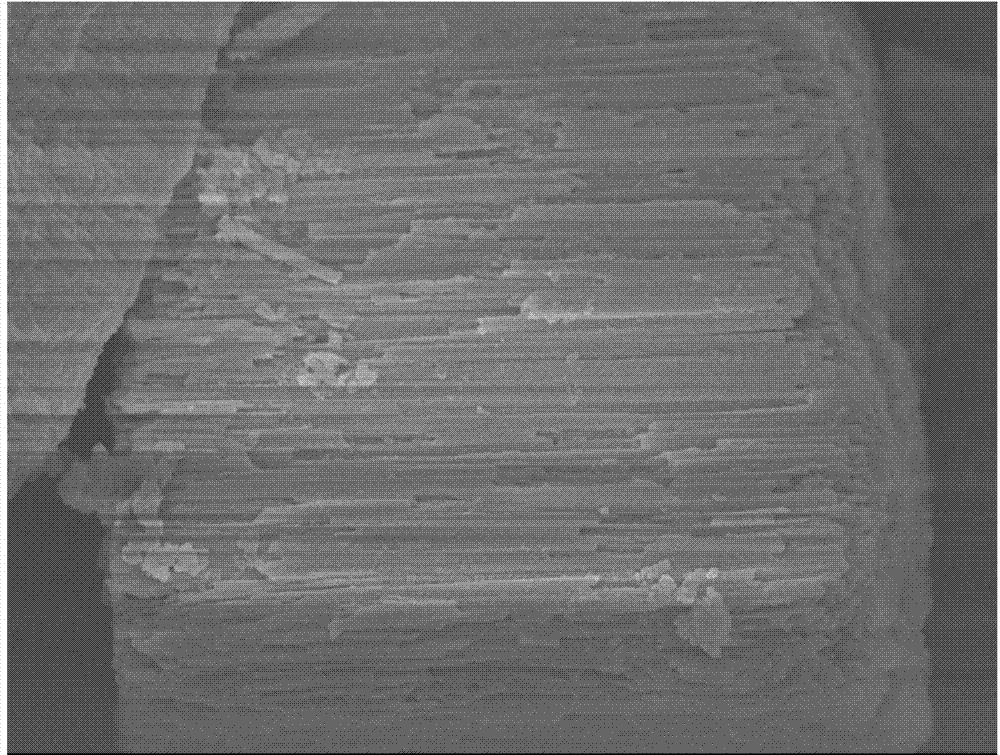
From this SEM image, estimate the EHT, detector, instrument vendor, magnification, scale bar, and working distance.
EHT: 8 kV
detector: SEI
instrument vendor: JEOL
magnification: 5 K X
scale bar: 1000 nm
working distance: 7.9 mm